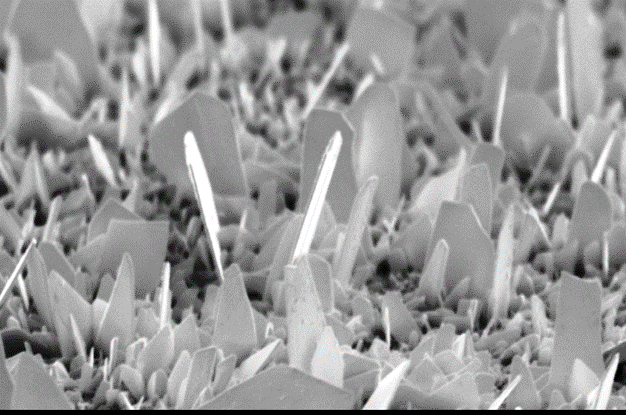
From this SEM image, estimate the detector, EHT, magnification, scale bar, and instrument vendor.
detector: SEI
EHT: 20 kV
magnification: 5 K X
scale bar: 5000 nm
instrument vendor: JEOL